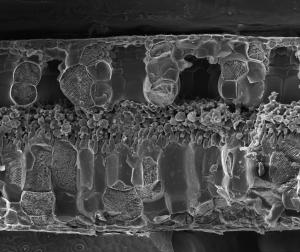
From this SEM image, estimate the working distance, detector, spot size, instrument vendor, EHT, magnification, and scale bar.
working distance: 10.4 mm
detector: ETD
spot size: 3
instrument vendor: FEI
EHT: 20 kV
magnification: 0.25 K X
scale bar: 500000 nm